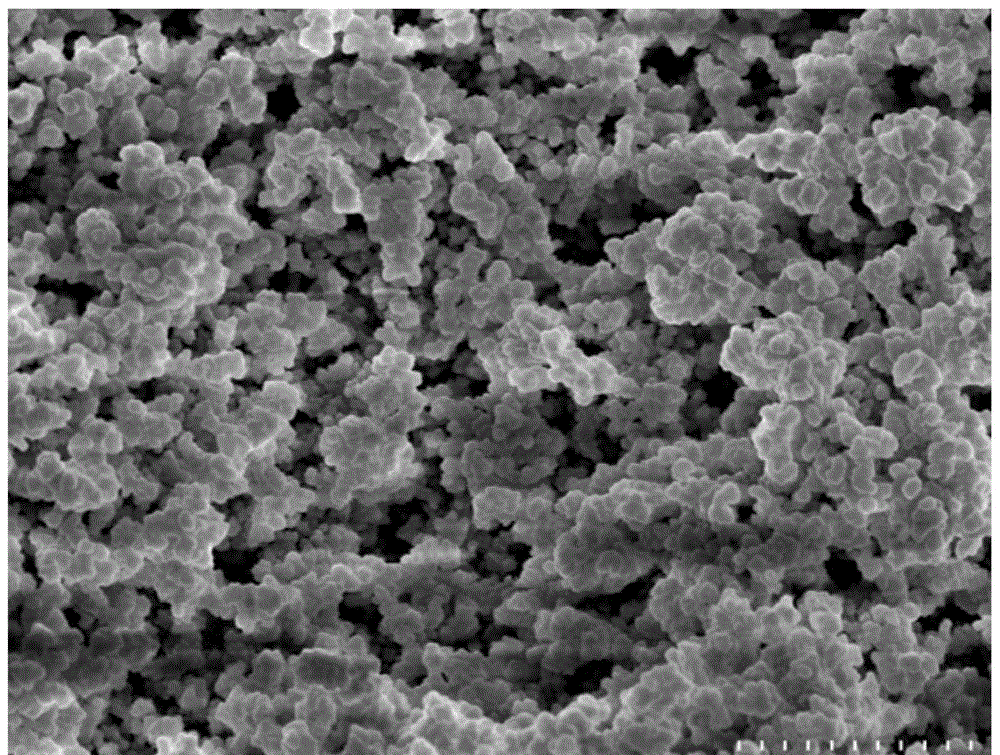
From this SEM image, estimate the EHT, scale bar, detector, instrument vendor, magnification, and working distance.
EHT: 5 kV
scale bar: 5000 nm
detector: SE(M)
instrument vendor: Hitachi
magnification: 6 K X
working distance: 10.3 mm